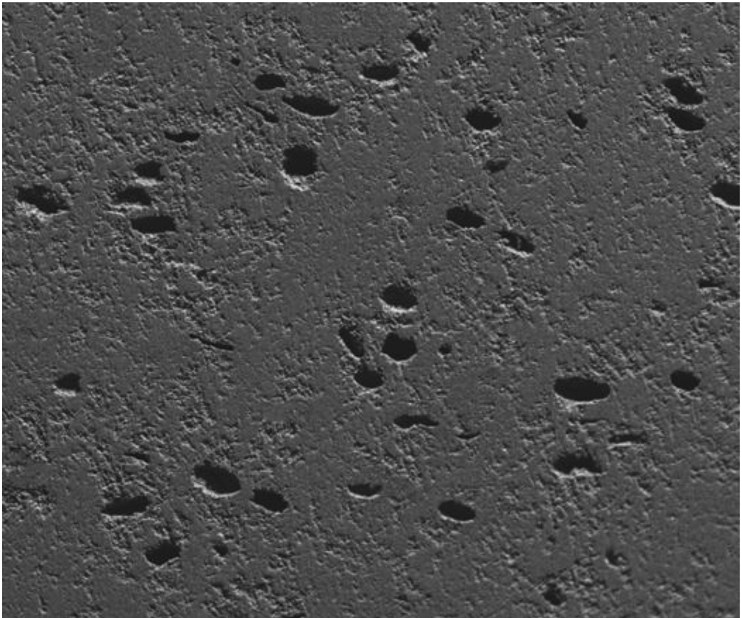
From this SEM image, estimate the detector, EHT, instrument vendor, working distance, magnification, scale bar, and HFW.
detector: ETD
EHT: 15 kV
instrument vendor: FEI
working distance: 8.4 mm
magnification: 0.18 K X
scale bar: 500000 nm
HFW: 1500 µm